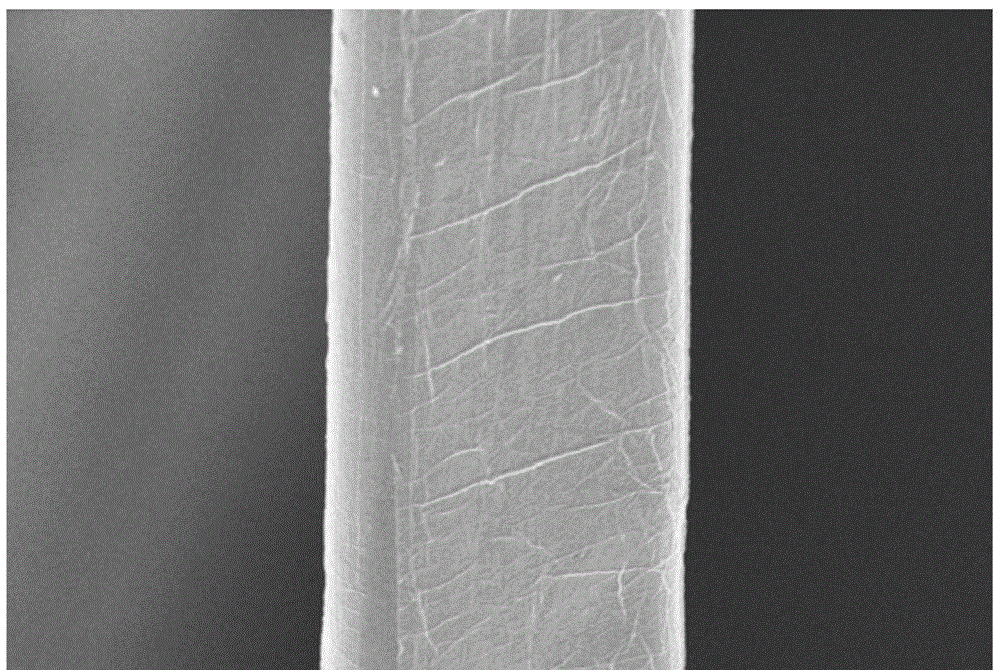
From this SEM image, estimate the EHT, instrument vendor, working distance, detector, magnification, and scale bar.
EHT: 5 kV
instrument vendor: Zeiss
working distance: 7.8 mm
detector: InLens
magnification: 20 K X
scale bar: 2000 nm